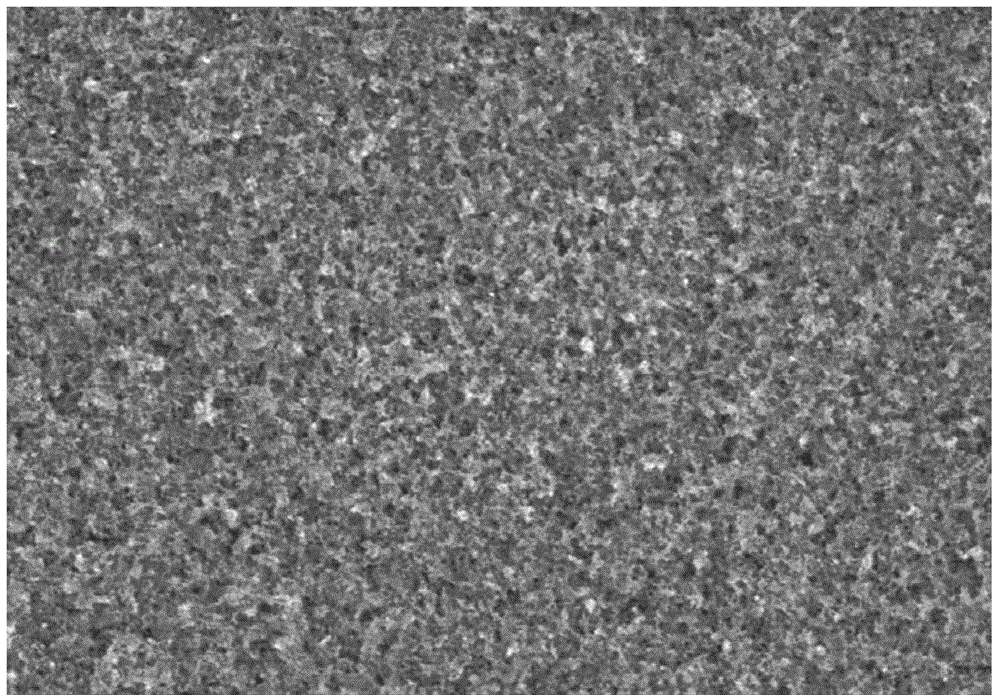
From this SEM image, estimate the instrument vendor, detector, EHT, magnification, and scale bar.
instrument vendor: Hitachi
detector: SE(U)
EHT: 4 kV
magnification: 40 K X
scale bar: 1000 nm